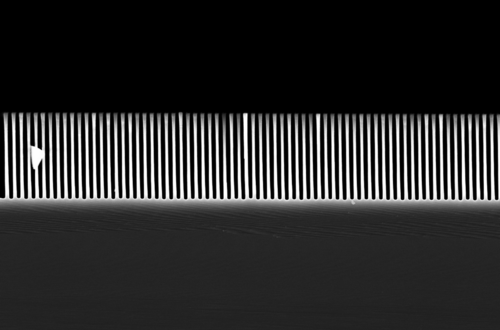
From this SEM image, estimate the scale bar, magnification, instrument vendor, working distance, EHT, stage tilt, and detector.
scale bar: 2000 nm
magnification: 10.87 K X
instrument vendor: Zeiss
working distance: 4.1 mm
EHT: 10 kV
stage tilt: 0°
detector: InLens